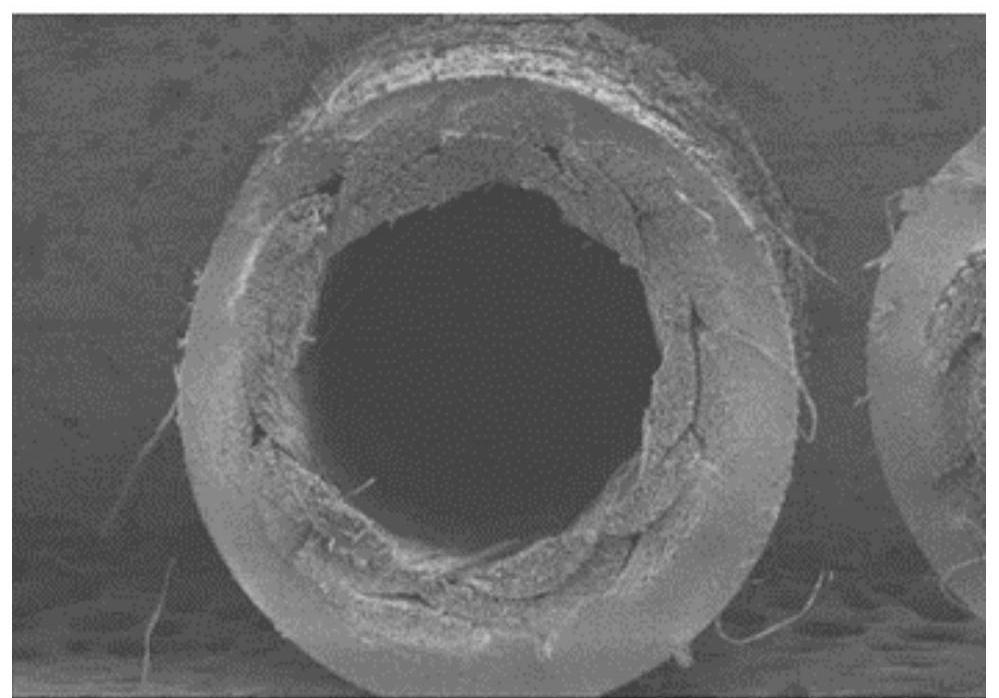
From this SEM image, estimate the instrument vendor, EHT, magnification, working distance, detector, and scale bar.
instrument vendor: Hitachi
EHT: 3 kV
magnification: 0.04 K X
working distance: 8.5 mm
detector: SE(M)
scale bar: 1000000 nm